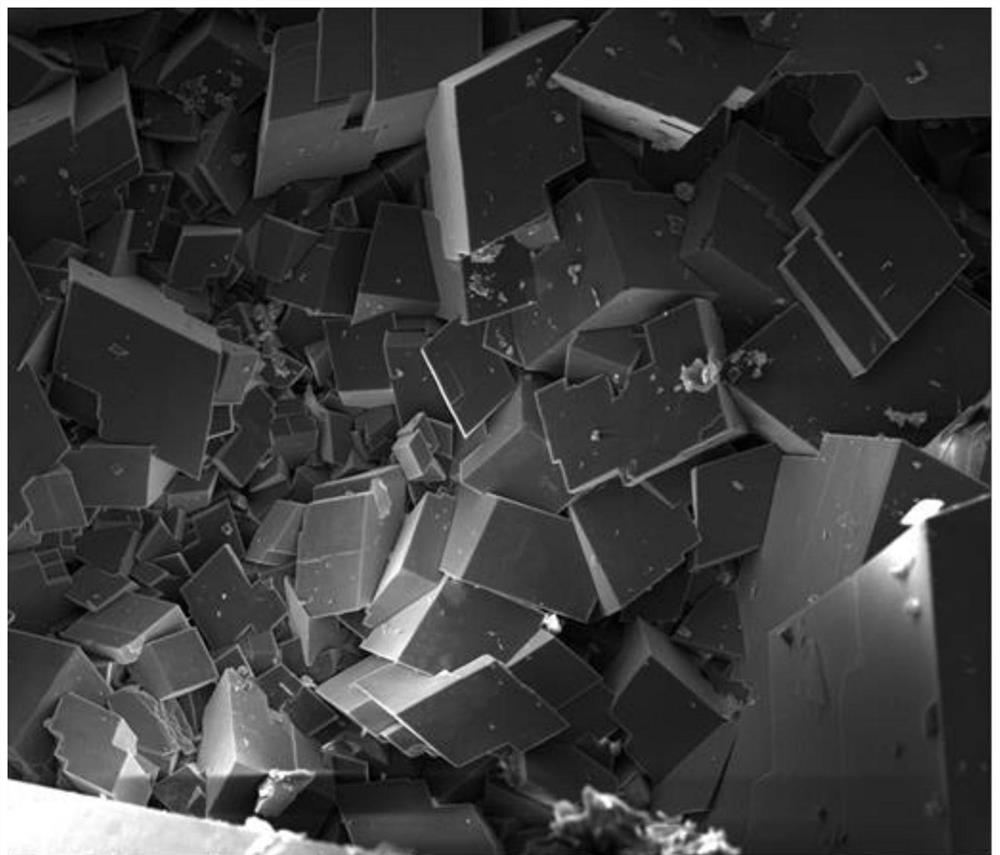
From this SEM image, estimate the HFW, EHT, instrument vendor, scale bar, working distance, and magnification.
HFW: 746 µm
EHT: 20 kV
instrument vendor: FEI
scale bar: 400000 nm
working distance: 14.3 mm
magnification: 0.4 K X